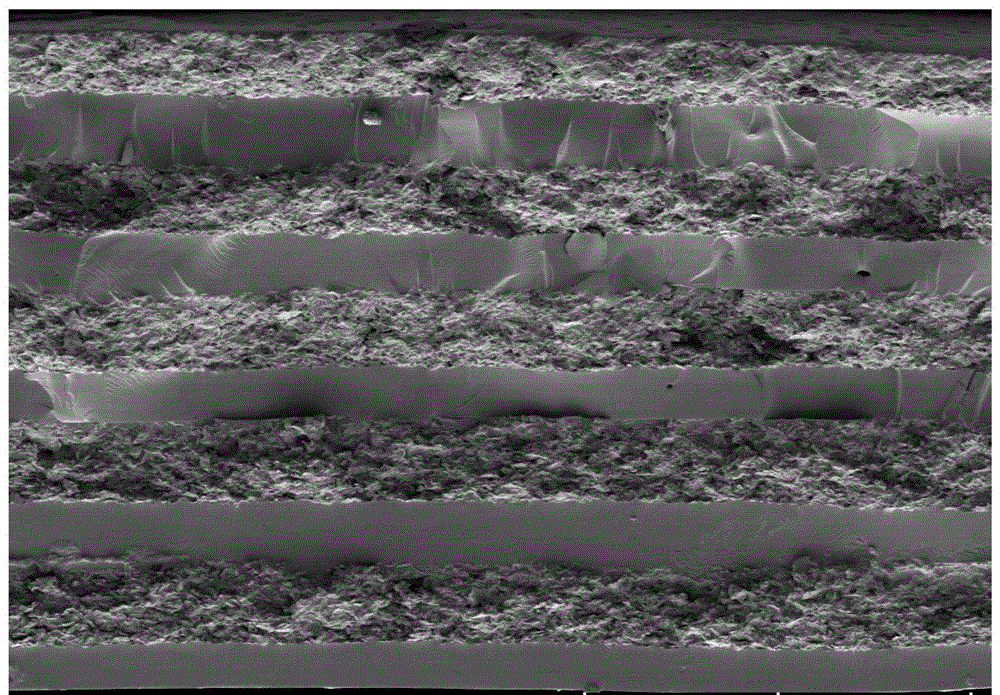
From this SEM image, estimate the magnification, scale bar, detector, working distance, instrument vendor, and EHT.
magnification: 0.1 K X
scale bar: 500000 nm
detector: LM(L)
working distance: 15.2 mm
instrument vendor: Hitachi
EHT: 5 kV